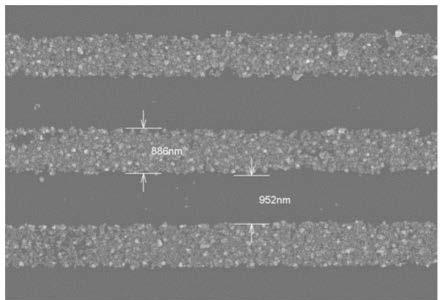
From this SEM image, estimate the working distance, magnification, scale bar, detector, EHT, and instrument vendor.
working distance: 8.1 mm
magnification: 15 K X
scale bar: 3000 nm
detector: SE(TUL)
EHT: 5 kV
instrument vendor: Hitachi